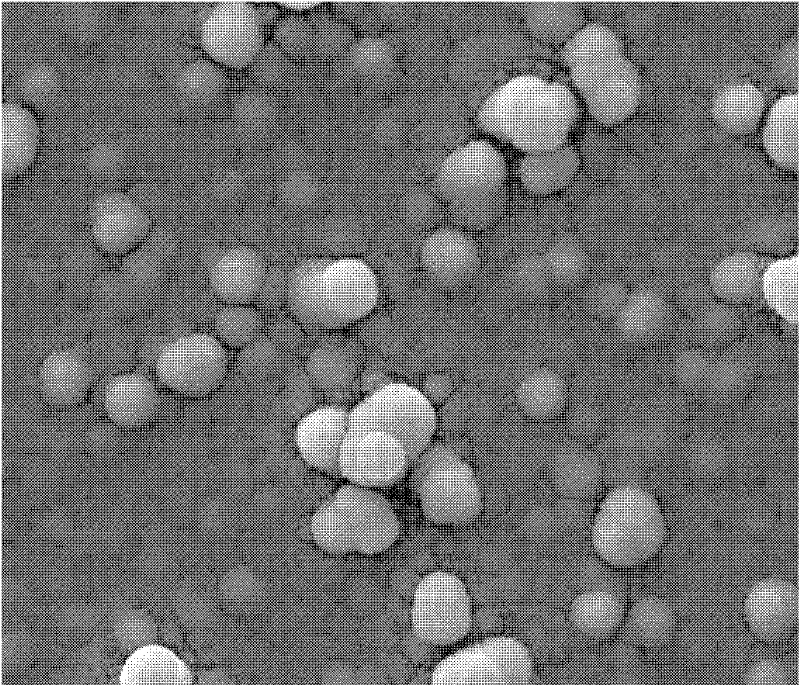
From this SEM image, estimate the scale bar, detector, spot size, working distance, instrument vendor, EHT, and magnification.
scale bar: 10000 nm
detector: ETD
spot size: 4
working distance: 10 mm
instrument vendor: FEI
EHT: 25 kV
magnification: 10 K X